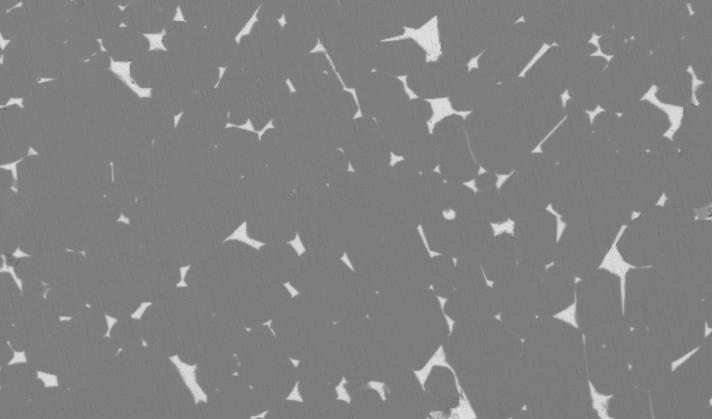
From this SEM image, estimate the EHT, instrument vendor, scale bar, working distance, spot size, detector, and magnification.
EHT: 20 kV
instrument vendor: FEI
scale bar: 50000 nm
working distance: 11 mm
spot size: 5.7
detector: BSE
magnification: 0.5 K X